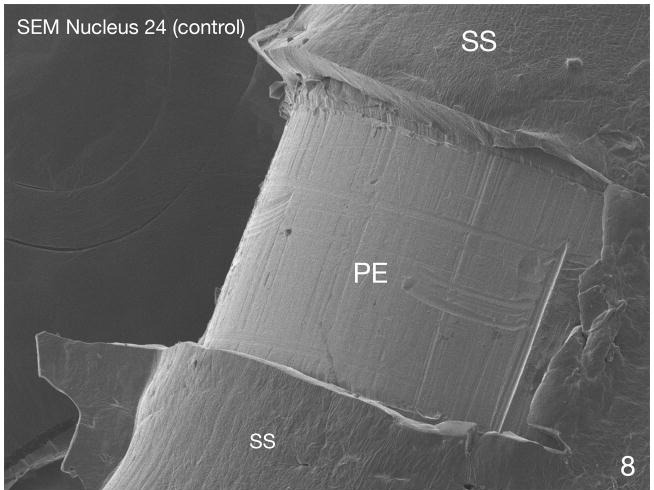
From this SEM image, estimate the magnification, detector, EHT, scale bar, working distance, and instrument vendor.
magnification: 0.25 K X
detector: LEI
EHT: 5 kV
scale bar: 100000 nm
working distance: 7.7 mm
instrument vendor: JEOL